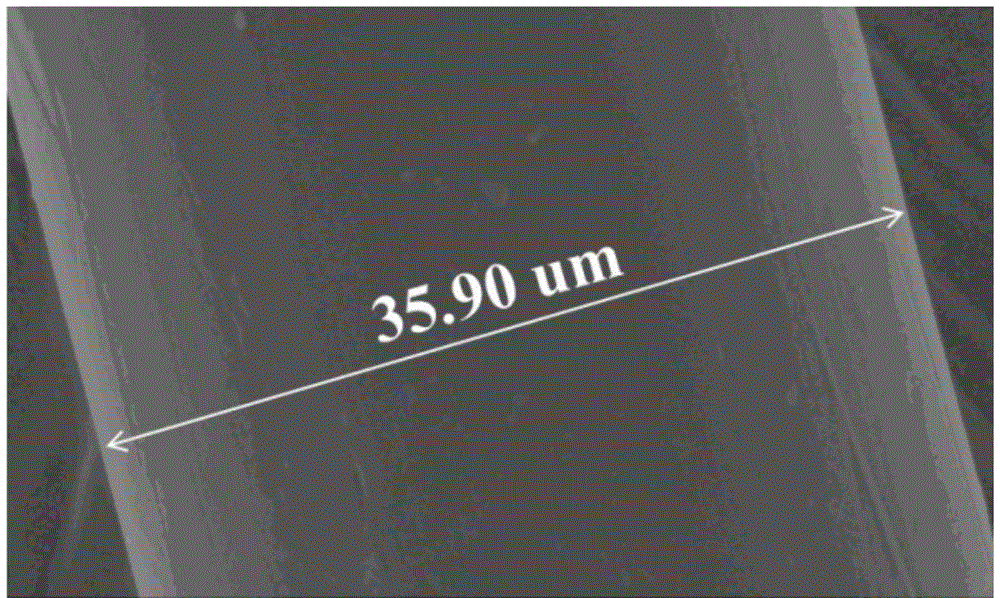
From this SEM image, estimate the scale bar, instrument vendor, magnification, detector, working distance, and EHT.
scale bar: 10000 nm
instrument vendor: Hitachi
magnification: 3 K X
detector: SE(U)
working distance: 9.5 mm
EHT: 5 kV